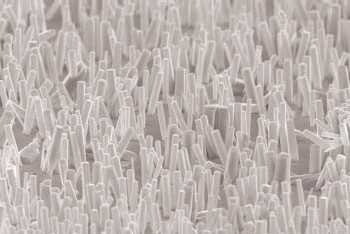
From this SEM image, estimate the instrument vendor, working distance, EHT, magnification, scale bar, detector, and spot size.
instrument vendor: FEI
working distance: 5 mm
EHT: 10 kV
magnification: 6.517 K X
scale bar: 2000 nm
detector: TLD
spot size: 3